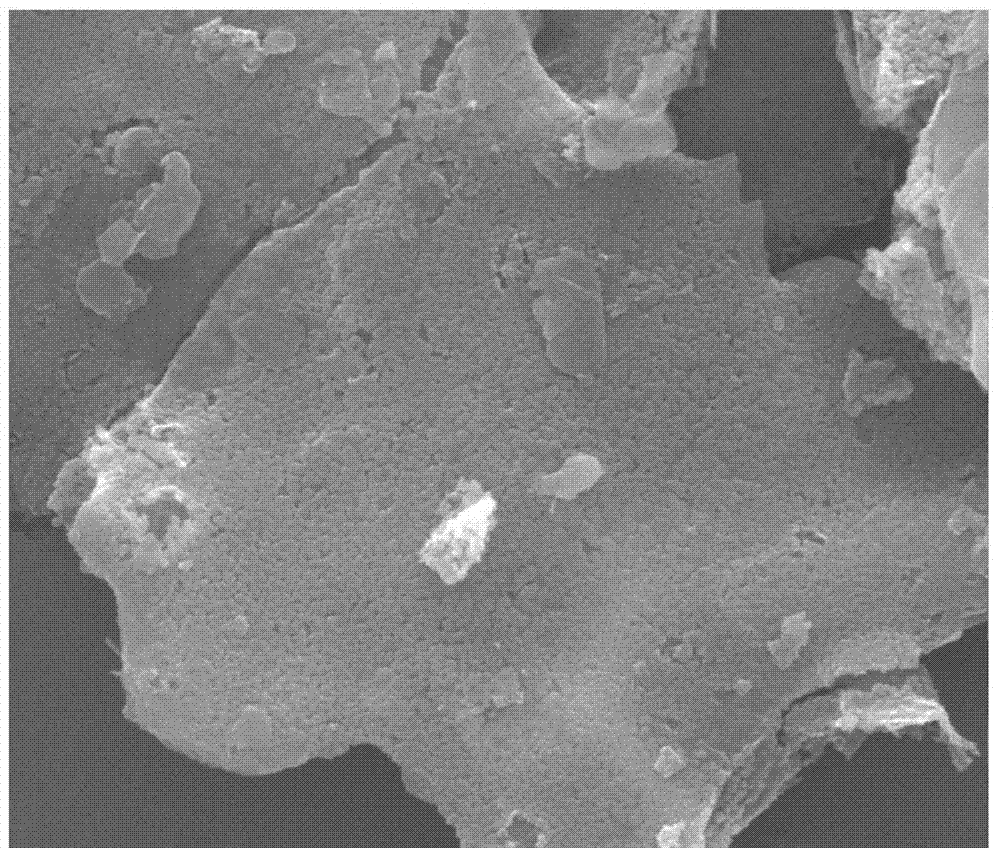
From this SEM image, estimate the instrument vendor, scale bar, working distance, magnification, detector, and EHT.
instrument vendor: FEI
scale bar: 5000 nm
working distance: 11.5 mm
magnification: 20 K X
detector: ETD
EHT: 20 kV